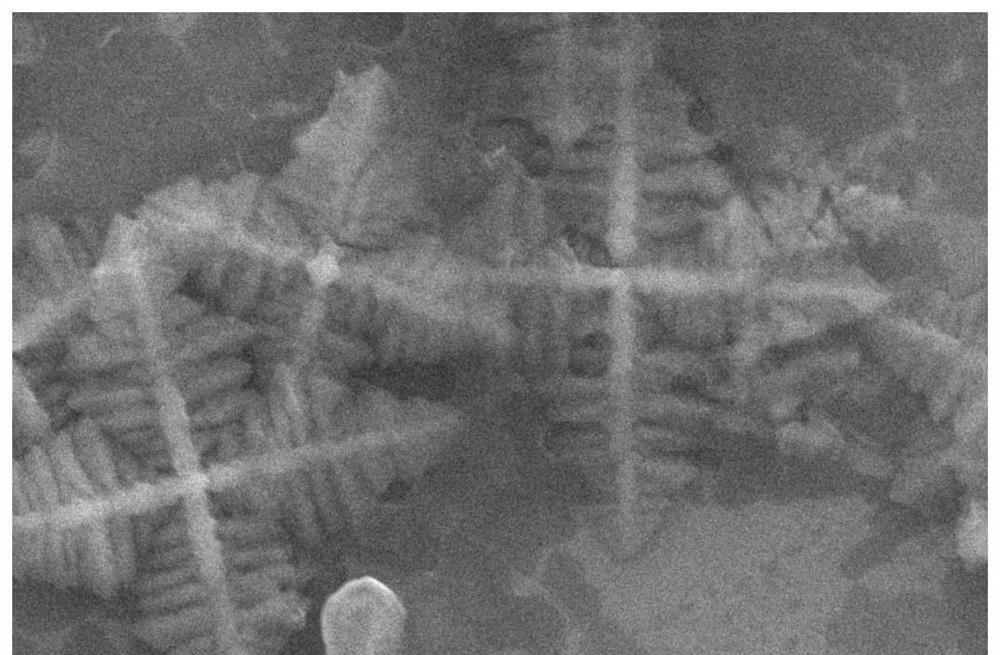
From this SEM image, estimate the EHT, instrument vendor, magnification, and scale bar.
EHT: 20 kV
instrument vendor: JEOL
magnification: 10 K X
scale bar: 1000 nm